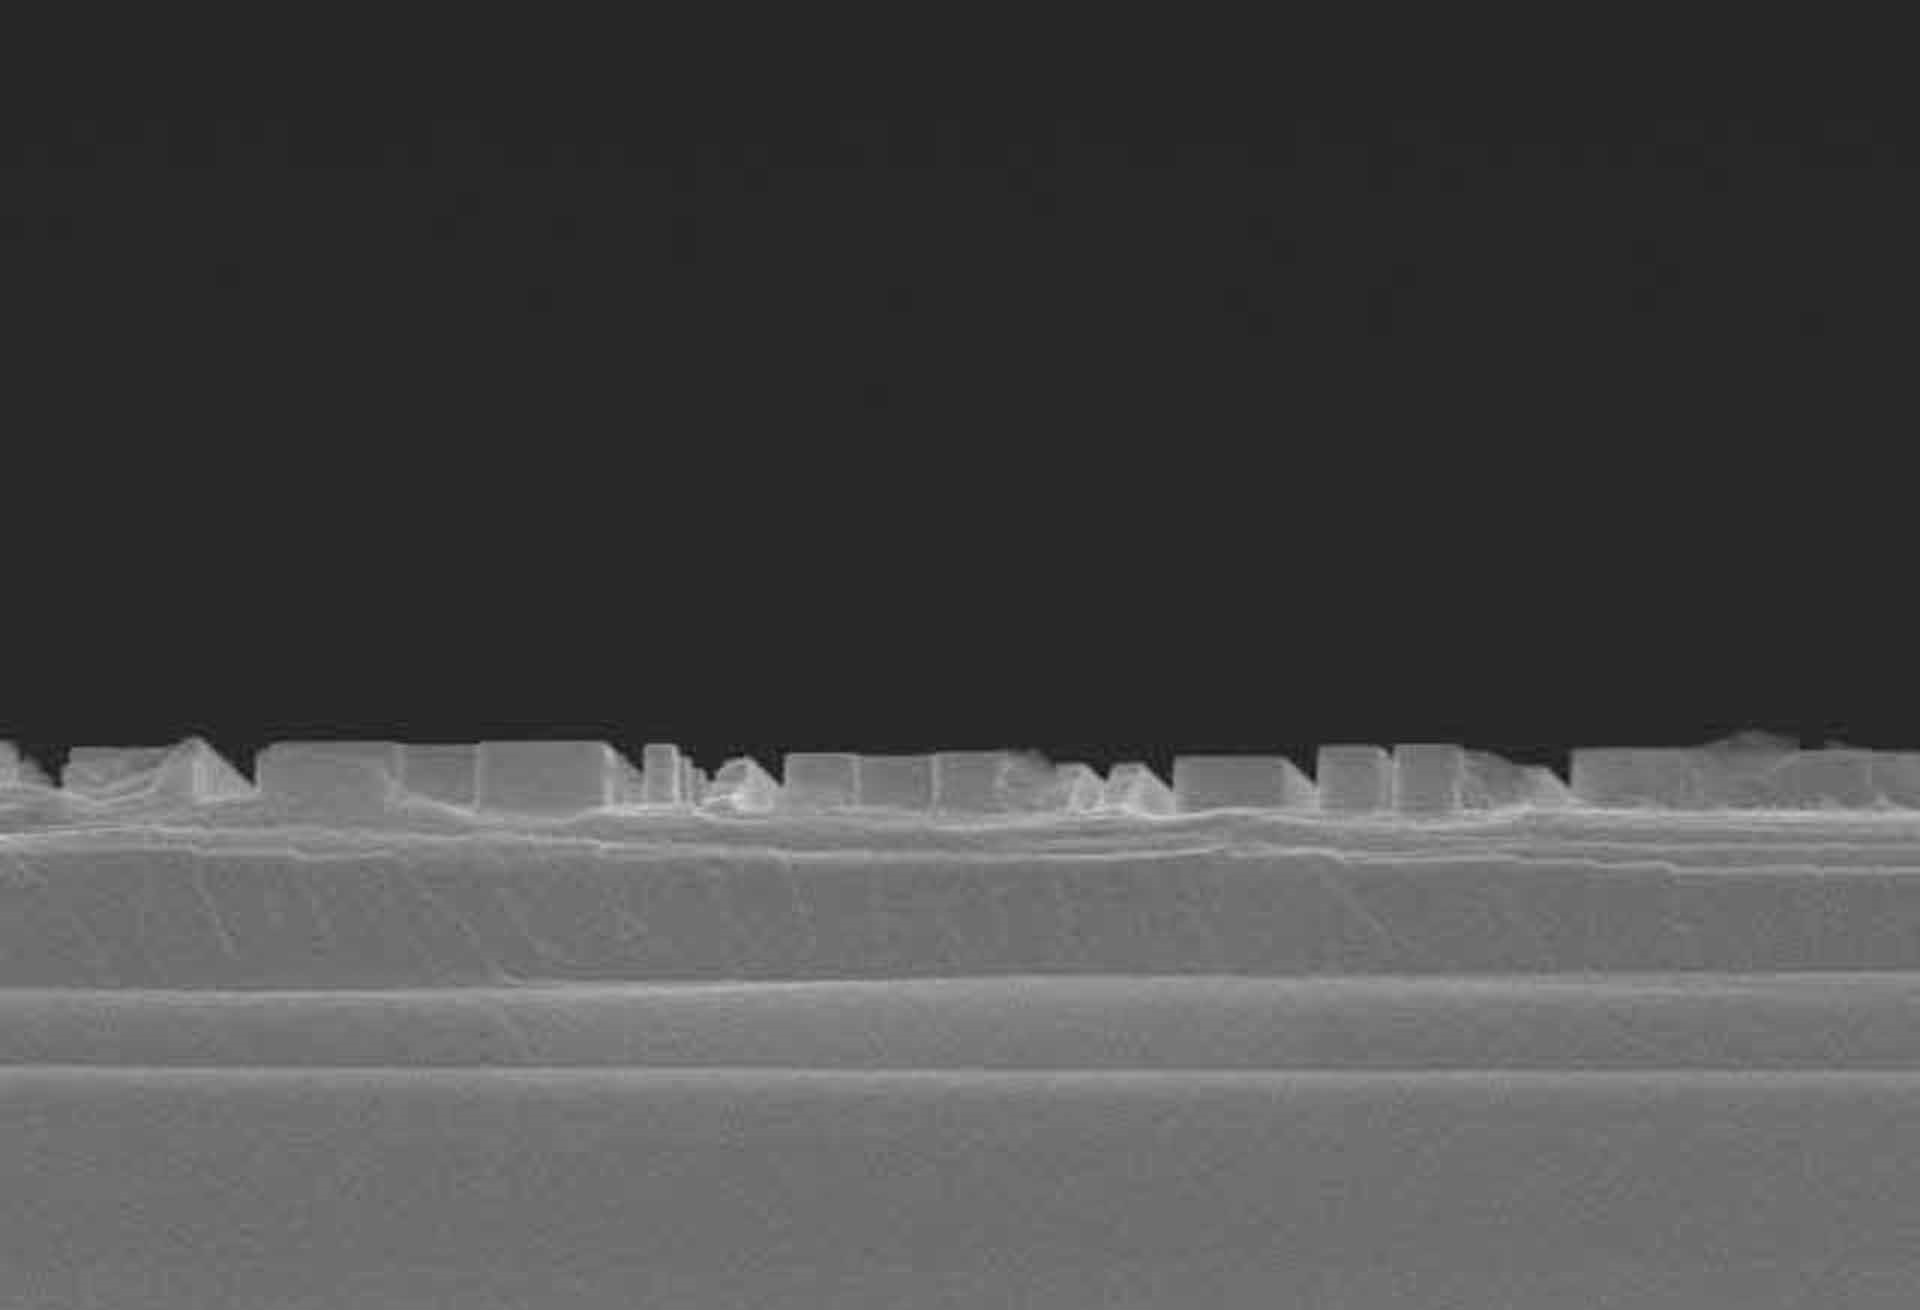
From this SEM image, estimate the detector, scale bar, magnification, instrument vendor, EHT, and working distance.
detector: SE(M)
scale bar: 3000 nm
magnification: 15 K X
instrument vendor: Hitachi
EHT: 15 kV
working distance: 15.8 mm